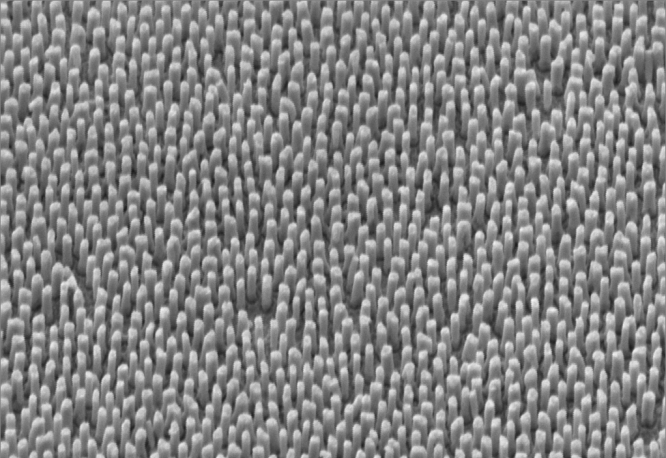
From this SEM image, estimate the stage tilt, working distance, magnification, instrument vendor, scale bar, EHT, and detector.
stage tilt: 45°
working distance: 6.1 mm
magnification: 73.54 K X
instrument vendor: Zeiss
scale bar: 200 nm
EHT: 4 kV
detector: SE2